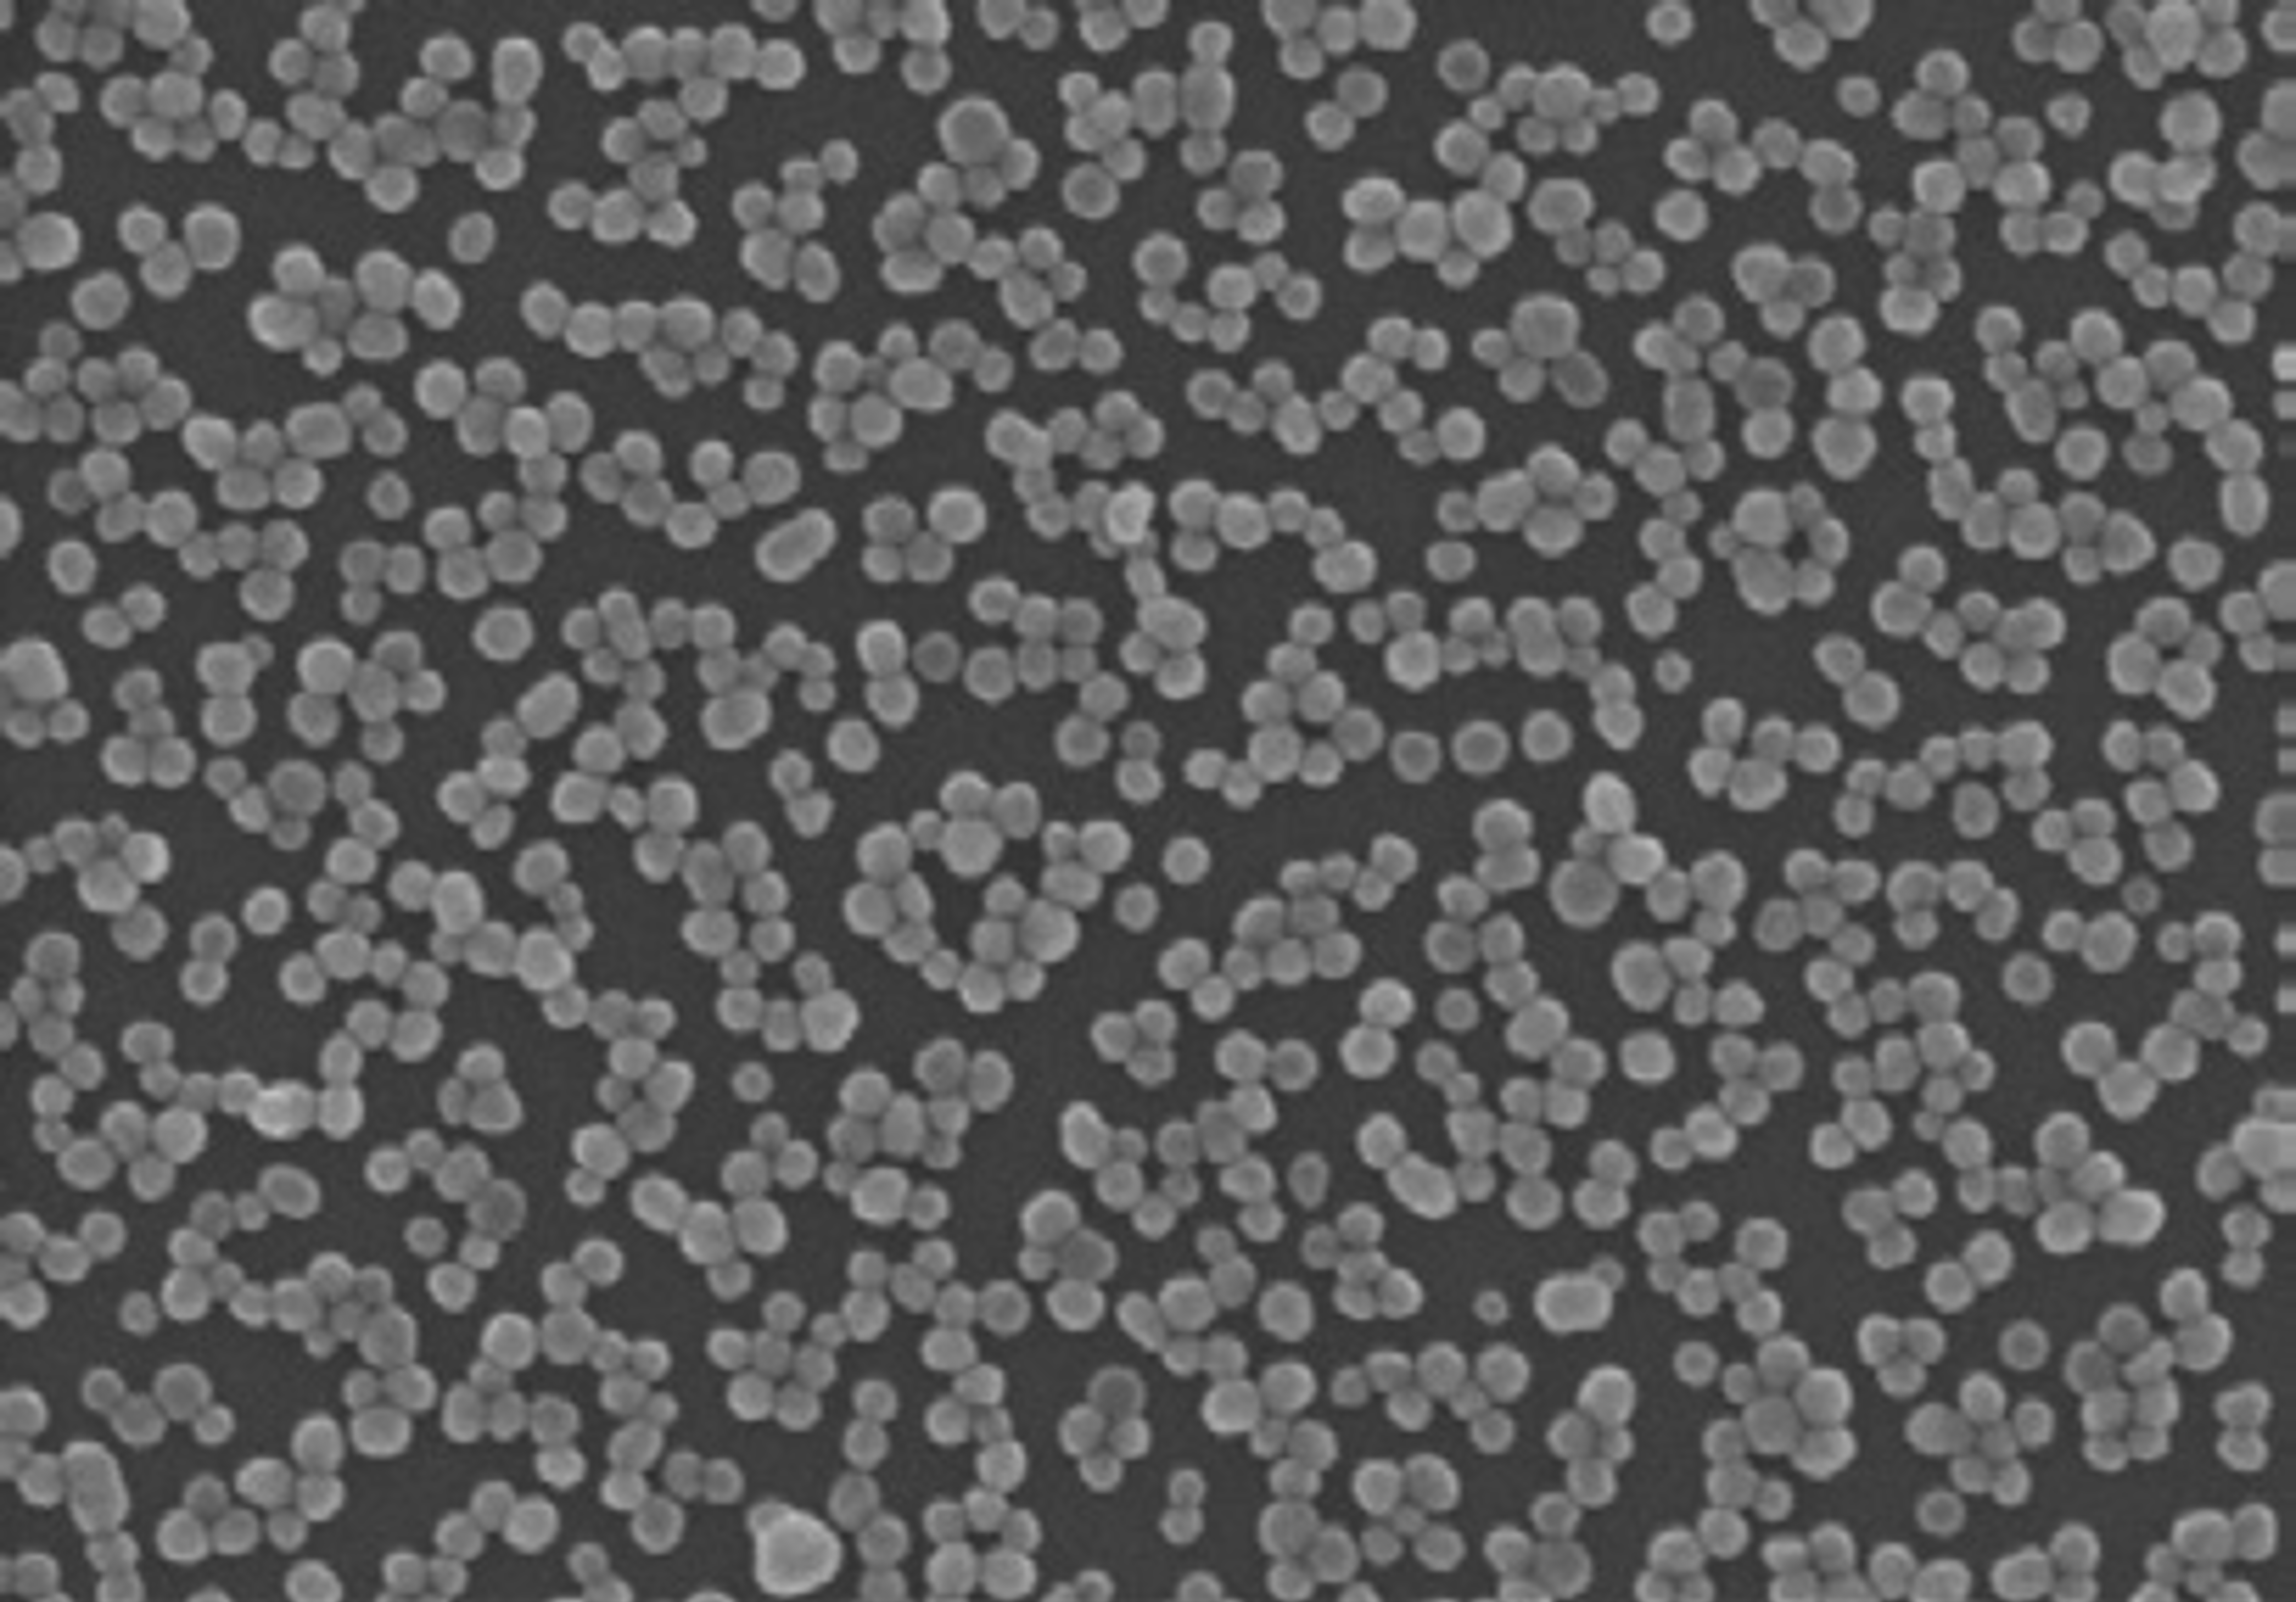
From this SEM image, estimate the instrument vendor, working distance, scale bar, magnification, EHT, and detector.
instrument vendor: Hitachi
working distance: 8.7 mm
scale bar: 1000 nm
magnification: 50 K X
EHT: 15 kV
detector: SE(U)